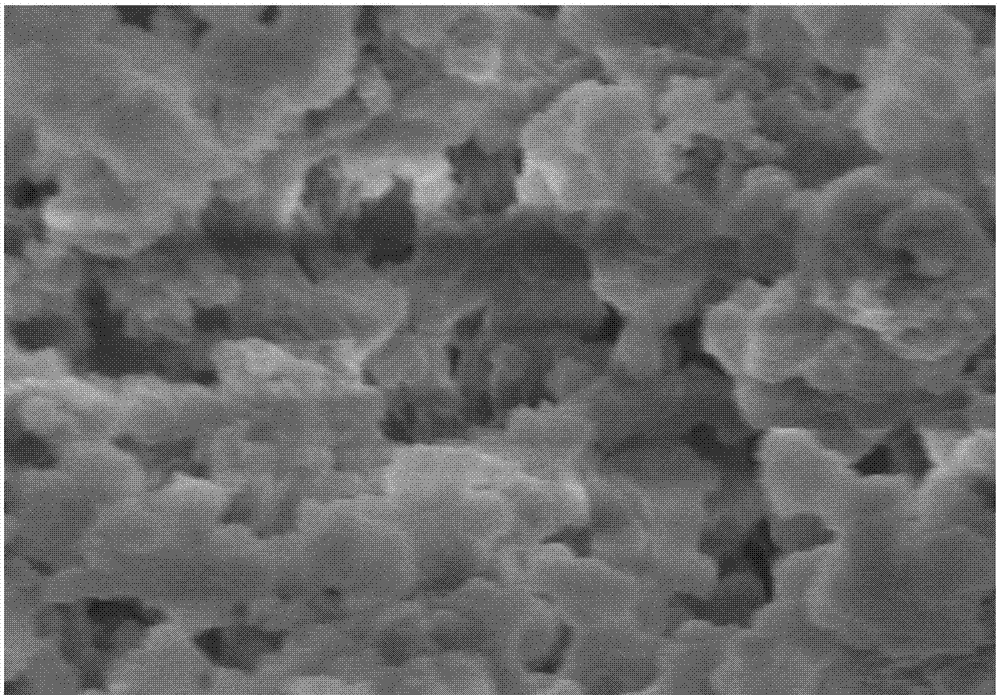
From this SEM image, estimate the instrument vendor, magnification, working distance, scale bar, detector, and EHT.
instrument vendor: Hitachi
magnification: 20 K X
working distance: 9.6 mm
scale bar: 2000 nm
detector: SE(M)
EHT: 5 kV